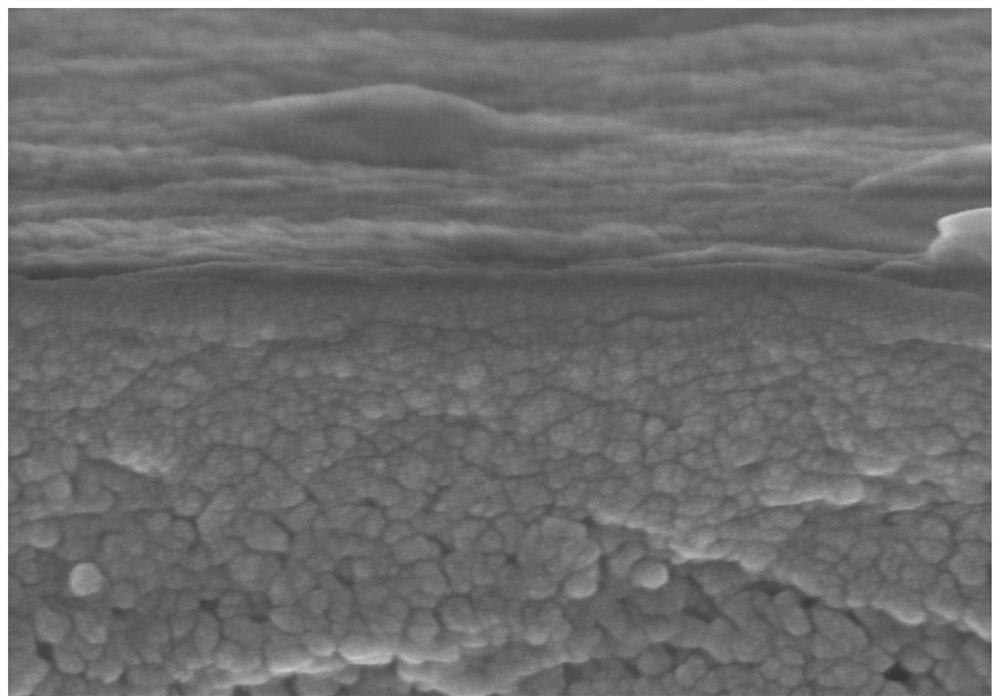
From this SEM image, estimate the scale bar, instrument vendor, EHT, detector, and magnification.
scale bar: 500 nm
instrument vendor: Hitachi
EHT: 8 kV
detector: SE(U)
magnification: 100 K X